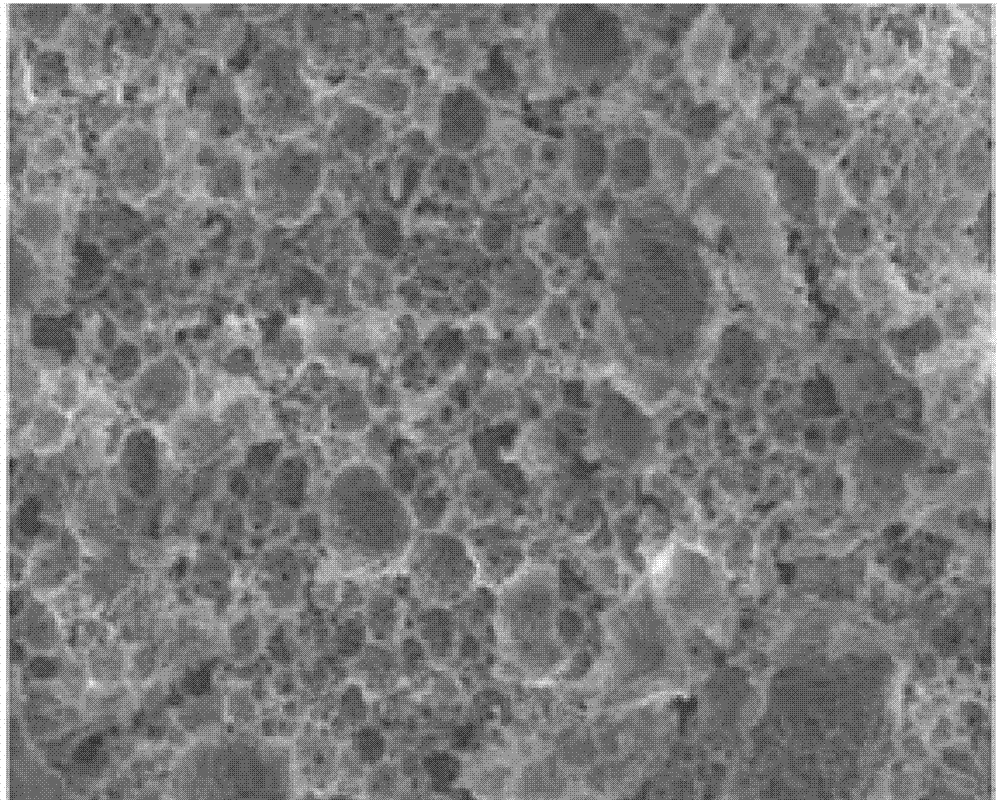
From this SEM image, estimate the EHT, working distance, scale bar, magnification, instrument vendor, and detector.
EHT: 5 kV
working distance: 5.8 mm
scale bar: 50000 nm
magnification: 1 K X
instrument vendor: Hitachi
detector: SE(L)